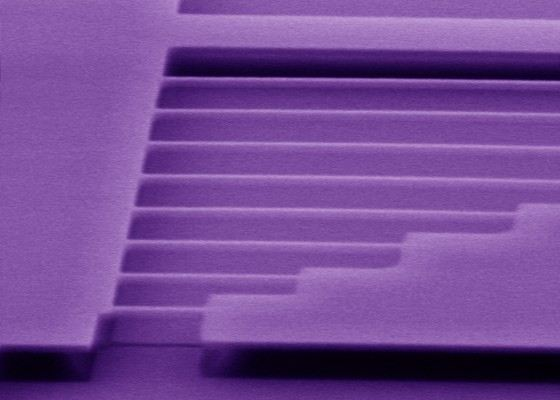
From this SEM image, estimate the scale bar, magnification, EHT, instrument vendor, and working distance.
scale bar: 2000 nm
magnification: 20 K X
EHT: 5 kV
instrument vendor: Hitachi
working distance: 5 mm